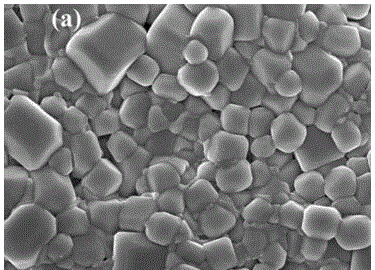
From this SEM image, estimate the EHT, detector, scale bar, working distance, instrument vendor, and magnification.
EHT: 15 kV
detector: SEI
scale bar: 1000 nm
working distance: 14.7 mm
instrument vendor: JEOL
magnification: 5 K X